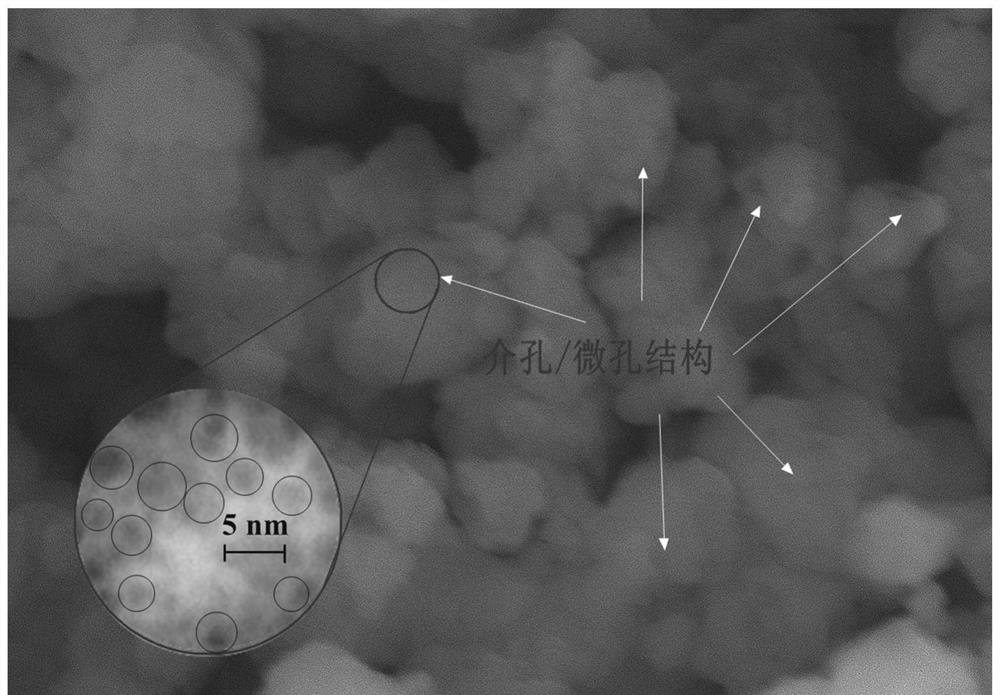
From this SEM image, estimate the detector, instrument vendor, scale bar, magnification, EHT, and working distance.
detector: SE(L)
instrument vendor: Hitachi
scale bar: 500 nm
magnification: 70 K X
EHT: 10 kV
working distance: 4.9 mm